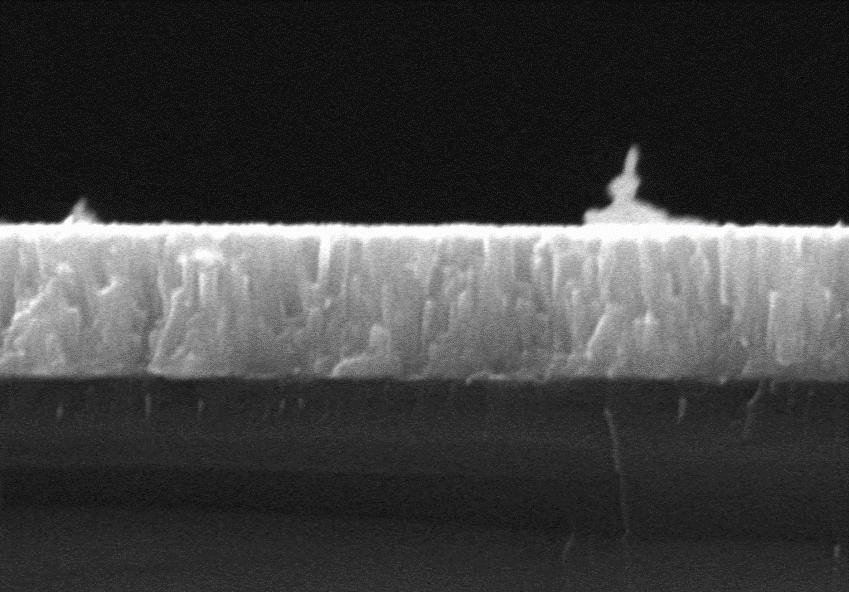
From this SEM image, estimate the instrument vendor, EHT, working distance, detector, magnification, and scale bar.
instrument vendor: Zeiss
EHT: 15 kV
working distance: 16.1 mm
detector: SE2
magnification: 25 K X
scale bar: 1000 nm